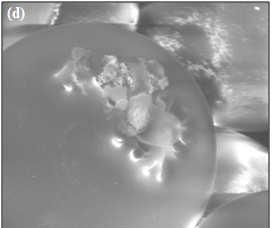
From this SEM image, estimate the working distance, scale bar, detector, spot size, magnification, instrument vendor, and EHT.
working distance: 11.1 mm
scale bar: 20000 nm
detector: LFD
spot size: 5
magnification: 1.8 K X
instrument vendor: FEI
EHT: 25 kV